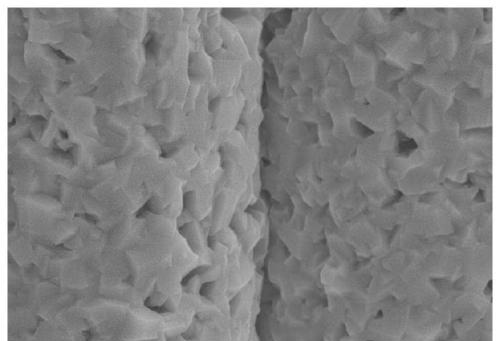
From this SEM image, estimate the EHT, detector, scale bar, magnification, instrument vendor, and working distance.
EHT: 15 kV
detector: SE(M)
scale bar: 5000 nm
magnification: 10 K X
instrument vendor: Hitachi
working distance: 11 mm